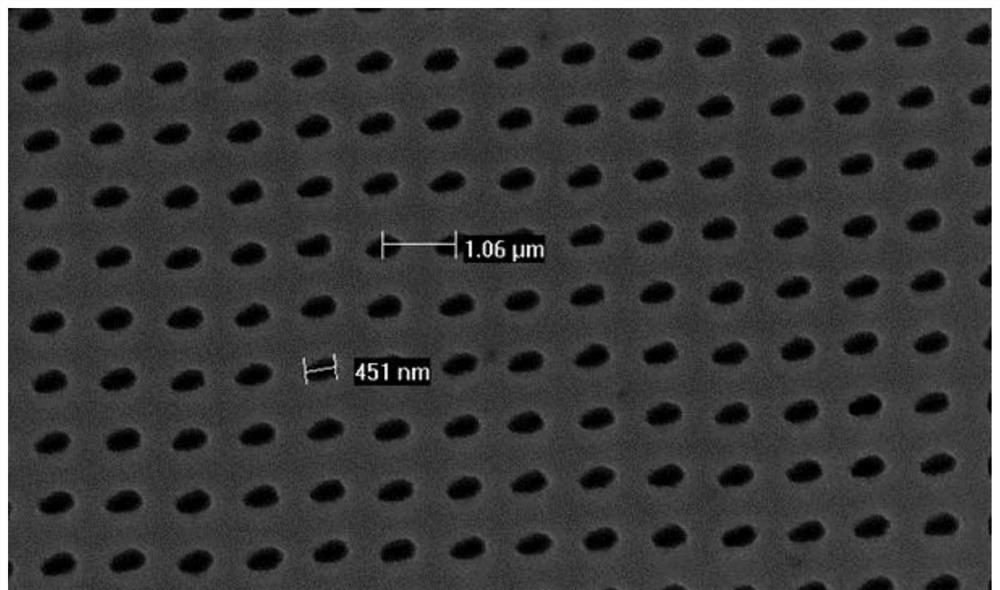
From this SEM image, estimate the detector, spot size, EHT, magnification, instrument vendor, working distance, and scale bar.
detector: TLD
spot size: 4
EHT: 20 kV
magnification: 20 K X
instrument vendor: FEI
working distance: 7.8 mm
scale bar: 2000 nm